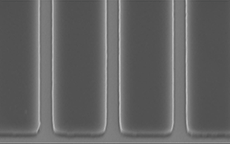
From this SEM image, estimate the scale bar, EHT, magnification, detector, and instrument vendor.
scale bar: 10000 nm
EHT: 15 kV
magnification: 2.5 K X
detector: SEI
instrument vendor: JEOL